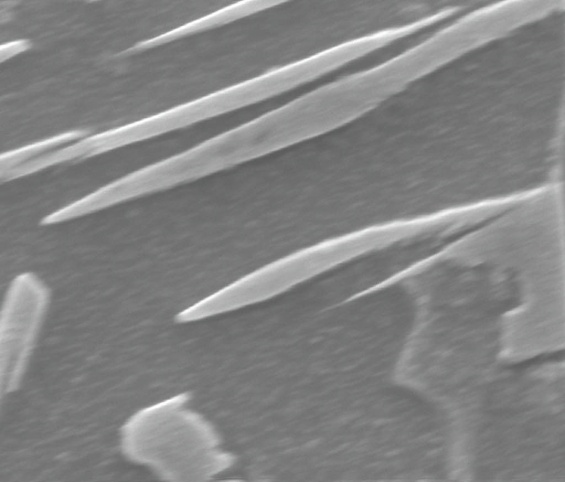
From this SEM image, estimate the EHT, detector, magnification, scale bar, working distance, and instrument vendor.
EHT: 15 kV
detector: SE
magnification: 60 K X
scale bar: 1000 nm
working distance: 10 mm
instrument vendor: FEI